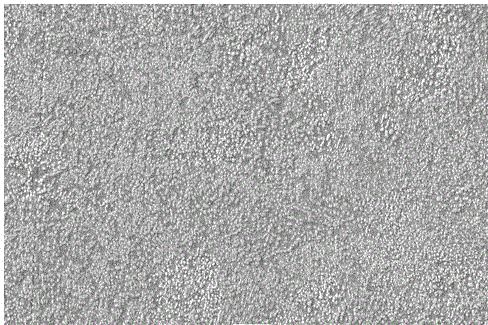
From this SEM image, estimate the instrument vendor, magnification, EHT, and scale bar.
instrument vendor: JEOL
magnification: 1 K X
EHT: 30 kV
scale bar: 10000 nm